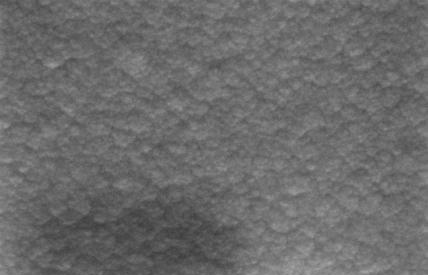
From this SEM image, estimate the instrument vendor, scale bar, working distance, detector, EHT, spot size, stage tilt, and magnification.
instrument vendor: Zeiss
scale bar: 1000 nm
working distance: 16.5 mm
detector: SE1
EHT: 20 kV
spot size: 300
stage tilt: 0°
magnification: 150 K X